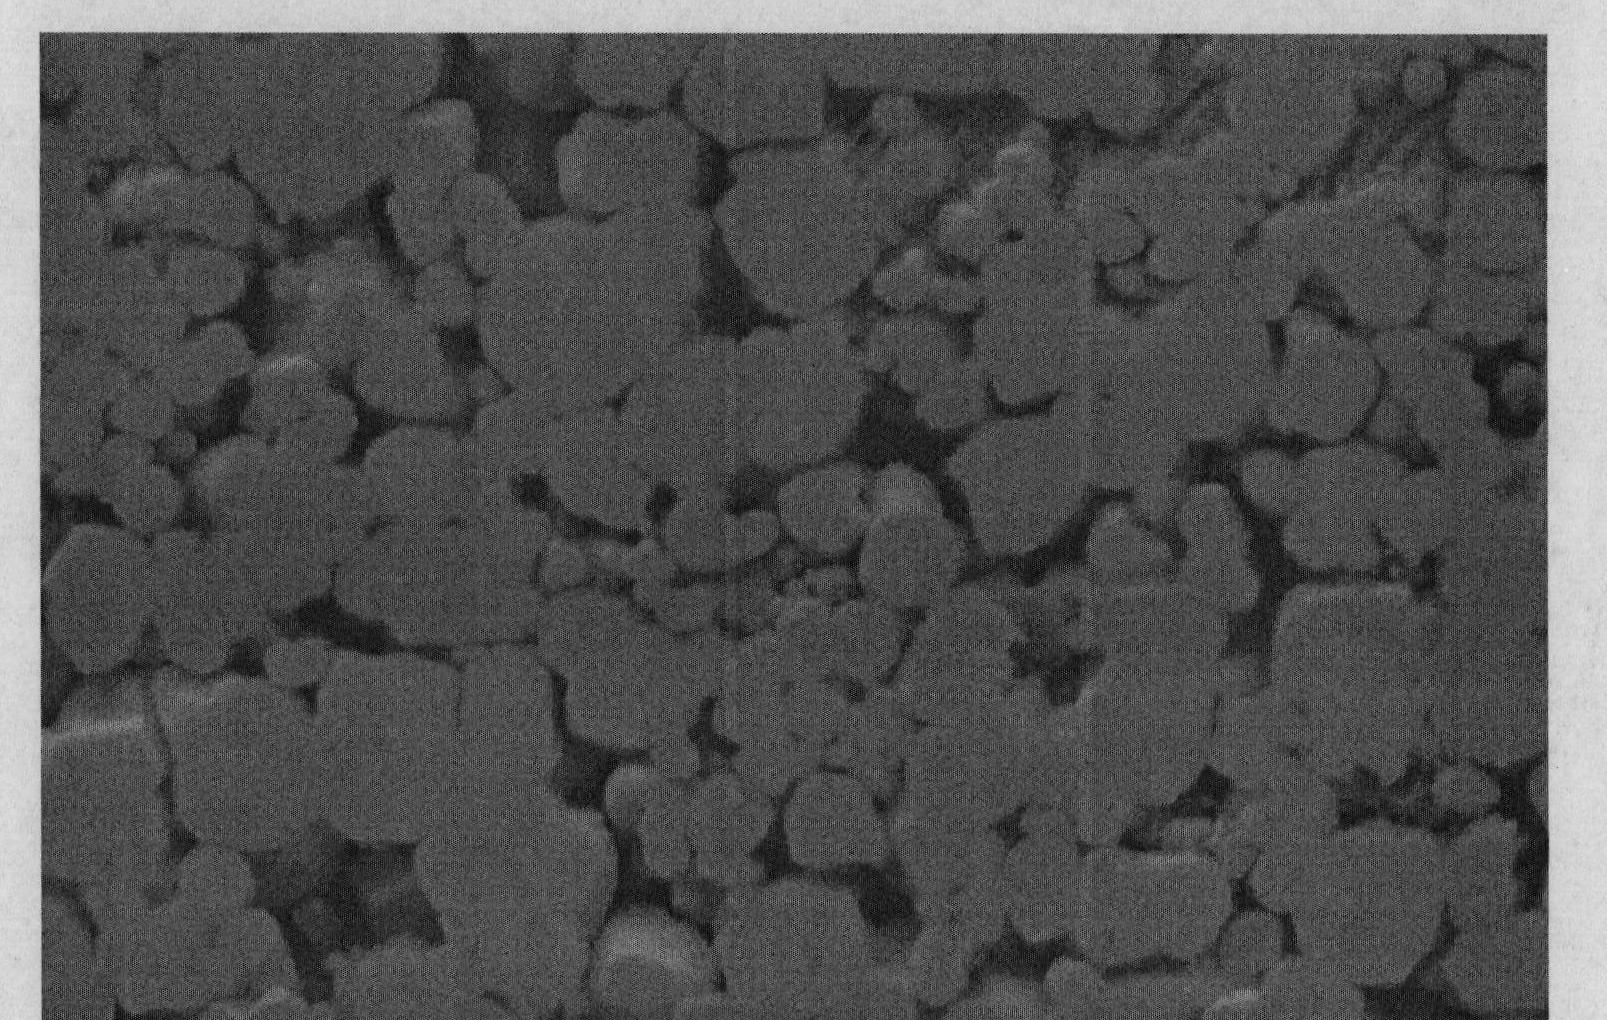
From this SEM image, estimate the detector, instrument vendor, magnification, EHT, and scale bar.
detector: SEI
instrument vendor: JEOL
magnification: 1.5 K X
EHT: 20 kV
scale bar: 10000 nm